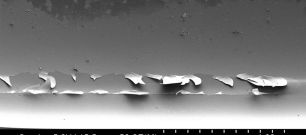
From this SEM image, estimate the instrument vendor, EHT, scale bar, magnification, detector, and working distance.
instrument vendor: Hitachi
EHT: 5 kV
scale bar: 1000000 nm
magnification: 0.05 K X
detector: SE(M)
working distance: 15.5 mm